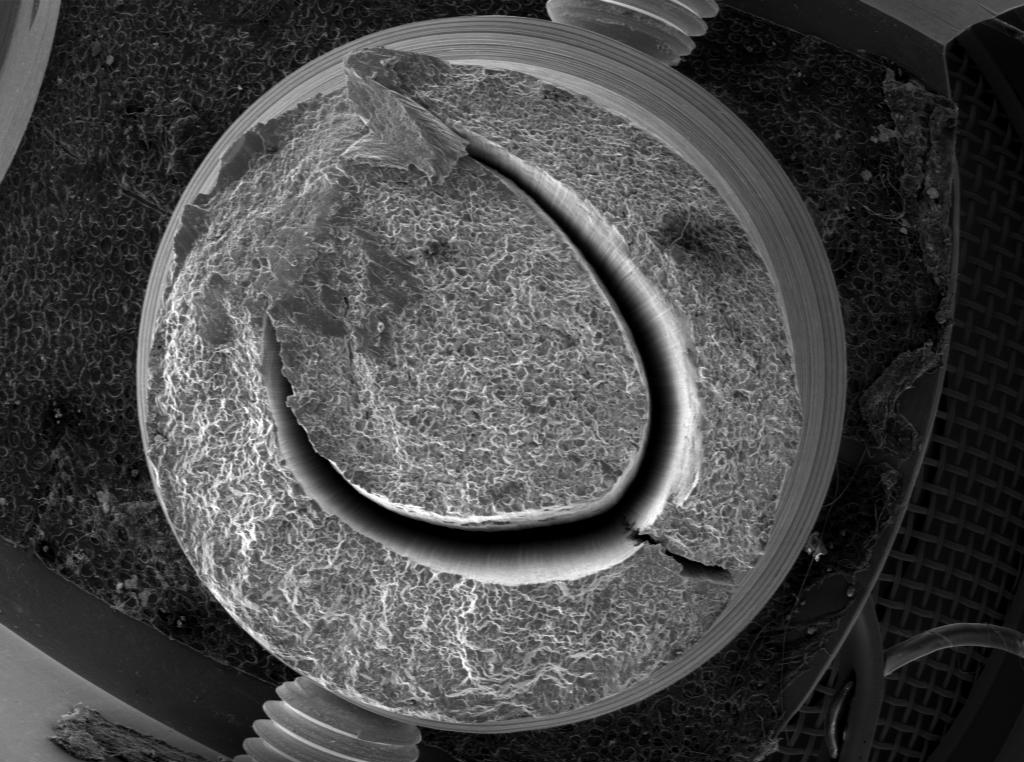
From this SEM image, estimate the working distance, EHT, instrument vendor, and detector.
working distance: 17.324 mm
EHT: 30 kV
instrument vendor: Tescan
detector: SE Detector